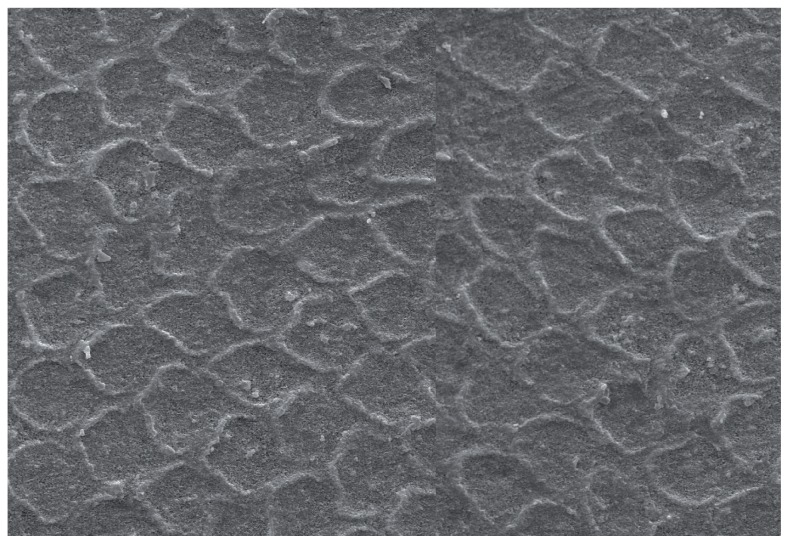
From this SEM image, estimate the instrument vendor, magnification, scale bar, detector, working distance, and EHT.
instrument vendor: Zeiss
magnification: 5 K X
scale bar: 2000 nm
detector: SE1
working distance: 12 mm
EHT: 20 kV